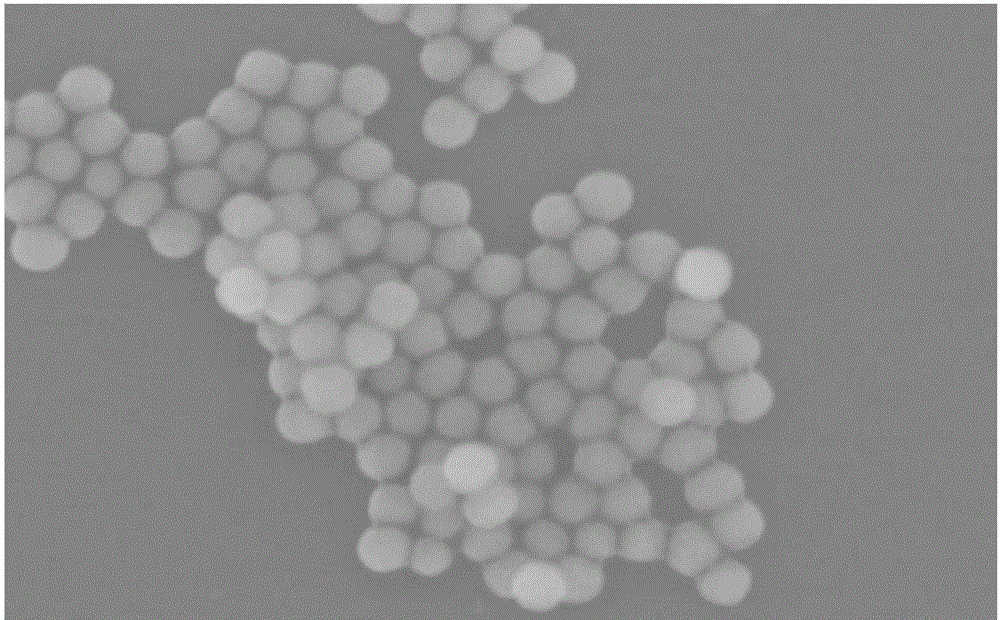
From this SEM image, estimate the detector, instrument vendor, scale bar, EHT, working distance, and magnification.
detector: SE(M)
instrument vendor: Hitachi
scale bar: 400 nm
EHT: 5 kV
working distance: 8.8 mm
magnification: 130 K X